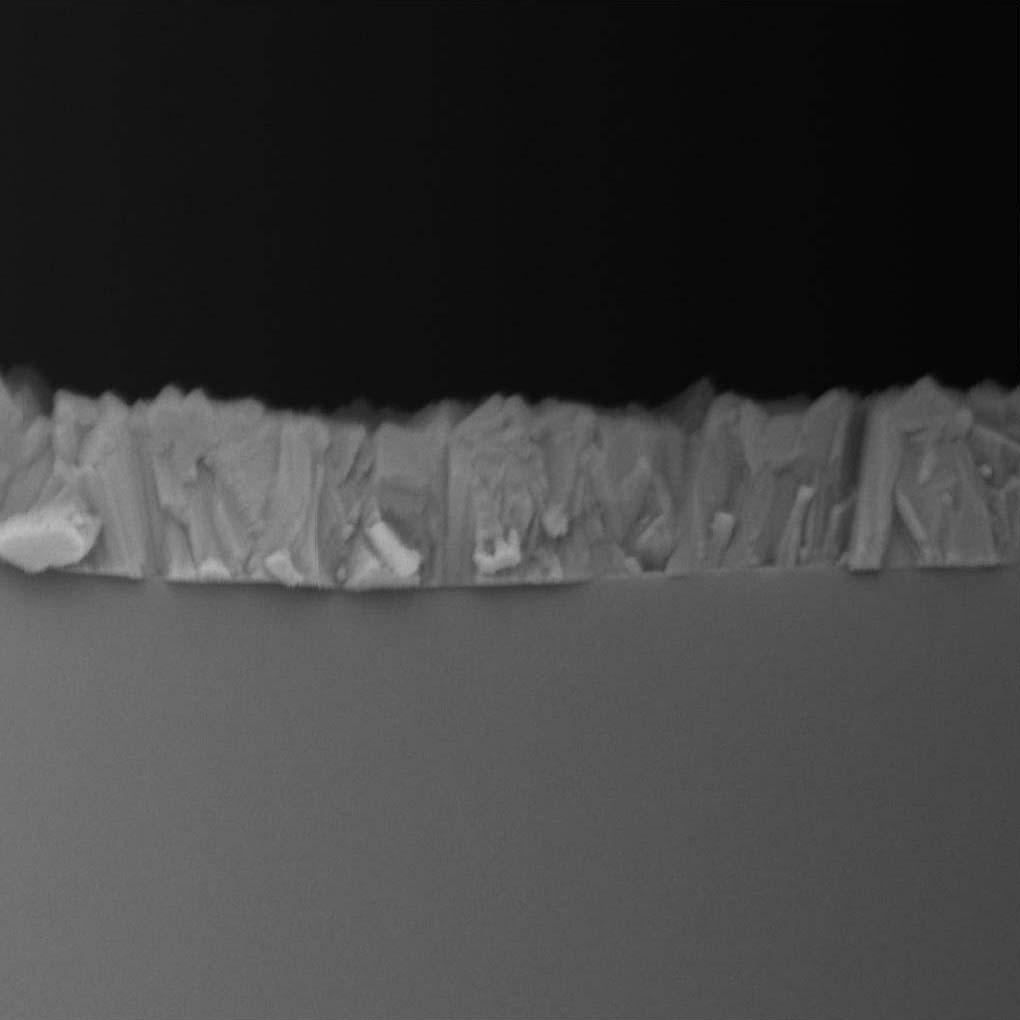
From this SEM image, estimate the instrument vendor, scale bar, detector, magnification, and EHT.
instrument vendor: Tescan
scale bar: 2000 nm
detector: BE Detector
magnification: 40 K X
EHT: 30 kV